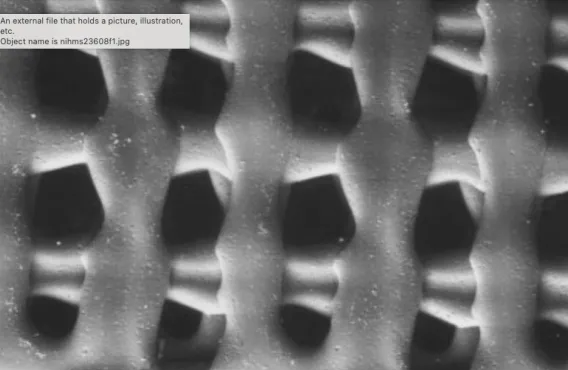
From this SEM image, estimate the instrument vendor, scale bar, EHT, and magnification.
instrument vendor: JEOL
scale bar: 2000000 nm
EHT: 25 kV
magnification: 0.025 K X